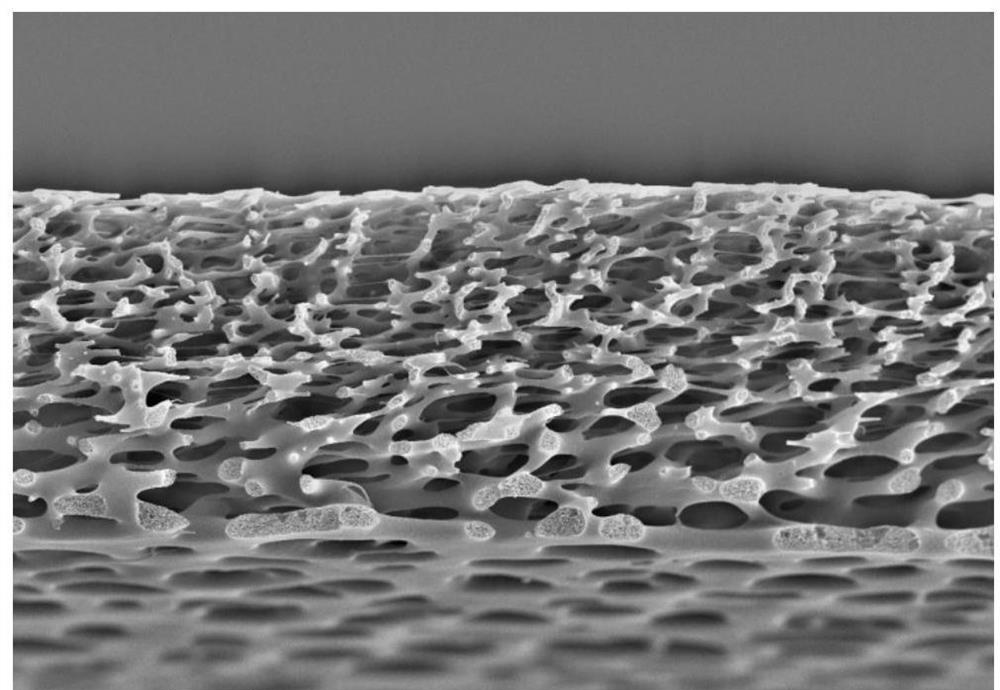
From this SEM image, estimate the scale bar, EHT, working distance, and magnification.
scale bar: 20000 nm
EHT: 20 kV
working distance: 6.4 mm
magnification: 0.5 K X